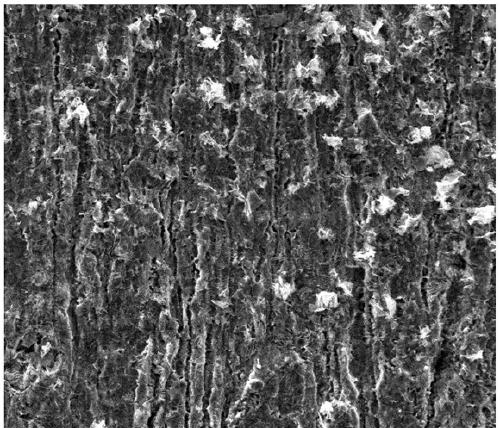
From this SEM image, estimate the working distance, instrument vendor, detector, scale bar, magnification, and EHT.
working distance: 10.3 mm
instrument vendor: FEI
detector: SE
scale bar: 50000 nm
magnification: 2 K X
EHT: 25 kV